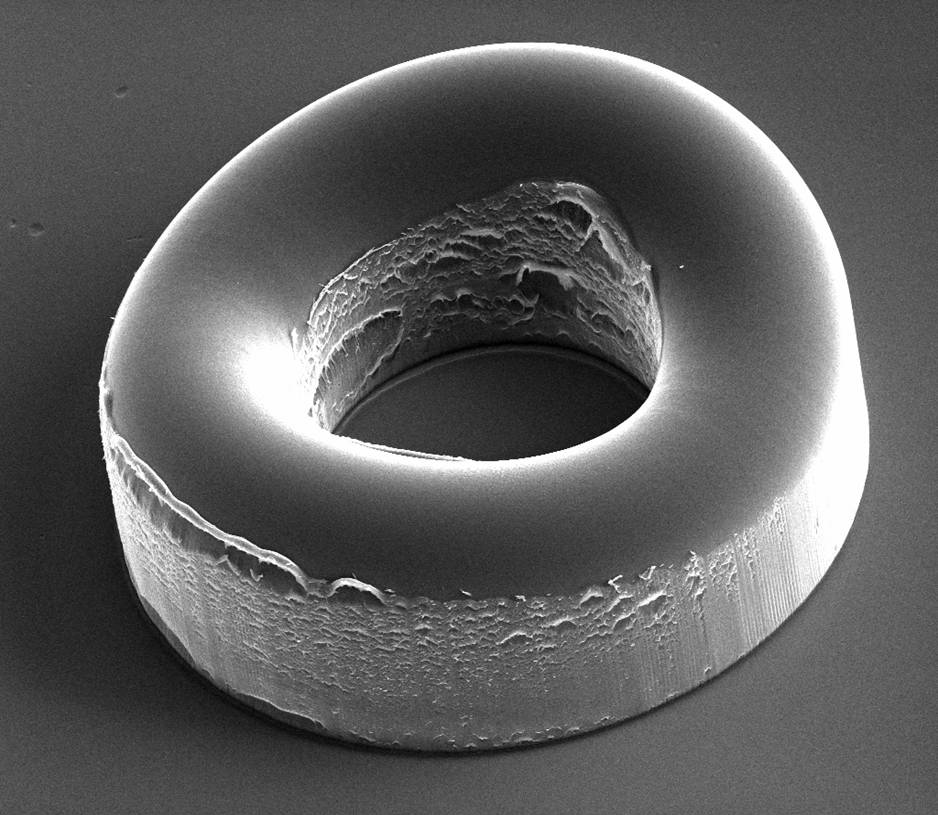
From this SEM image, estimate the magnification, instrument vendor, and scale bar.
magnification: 1.2 K X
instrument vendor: FEI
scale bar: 200000 nm